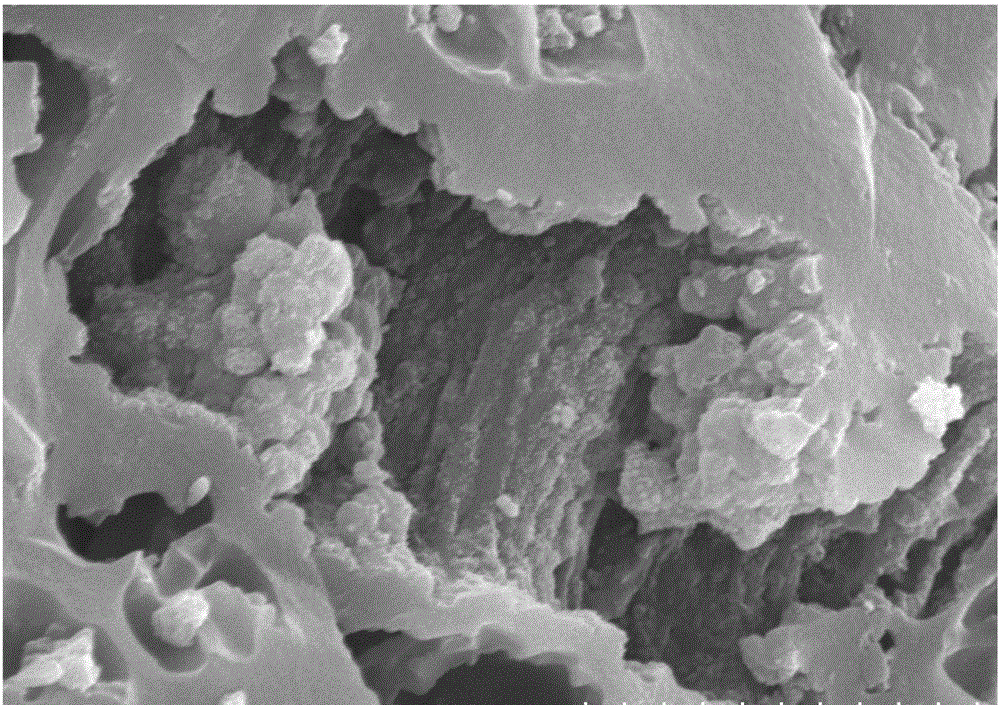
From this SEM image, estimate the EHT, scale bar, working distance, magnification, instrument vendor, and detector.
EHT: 15 kV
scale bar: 5000 nm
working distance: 8.3 mm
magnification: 10 K X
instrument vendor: Hitachi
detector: SE